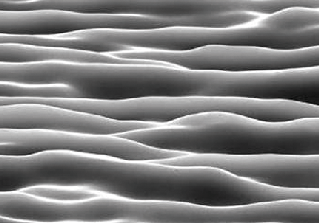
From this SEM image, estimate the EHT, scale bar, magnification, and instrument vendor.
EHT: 25 kV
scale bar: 20000 nm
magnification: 2.5 K X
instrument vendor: JEOL